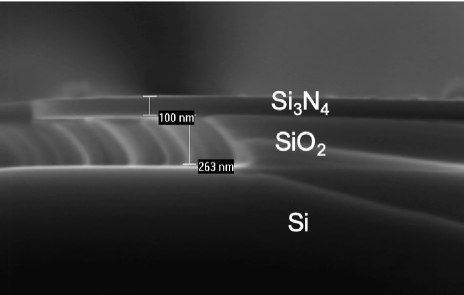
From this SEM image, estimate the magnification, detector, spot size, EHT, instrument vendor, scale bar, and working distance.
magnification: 100 K X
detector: SE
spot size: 3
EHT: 12 kV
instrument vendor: FEI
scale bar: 500 nm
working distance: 8.9 mm